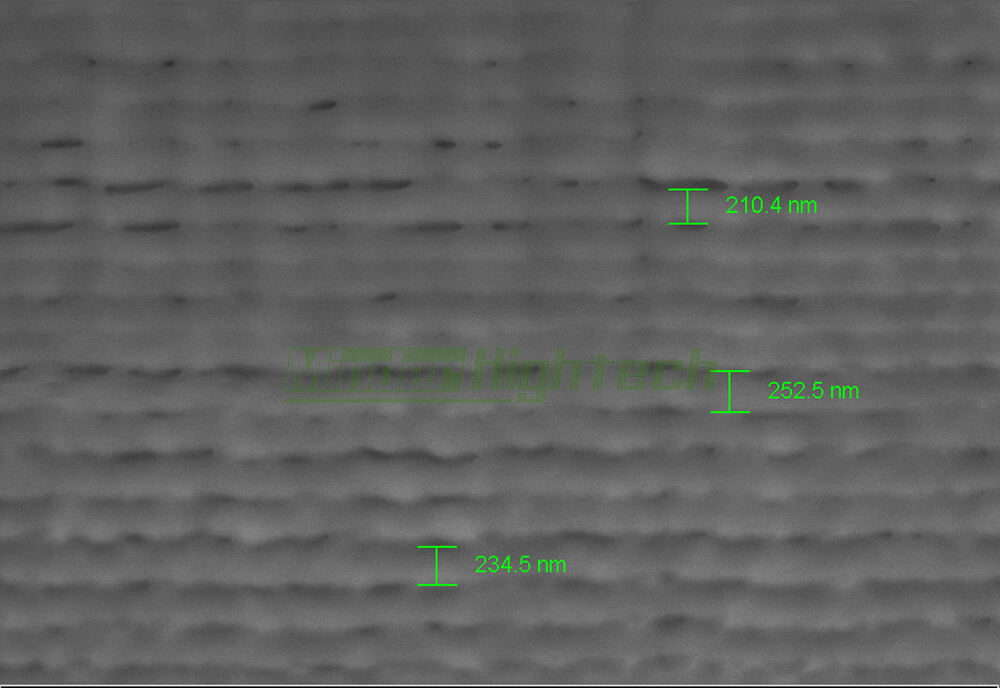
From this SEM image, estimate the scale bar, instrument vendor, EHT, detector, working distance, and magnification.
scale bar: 400 nm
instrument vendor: Zeiss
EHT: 6 kV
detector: SE2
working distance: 9.2 mm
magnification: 18.57 K X